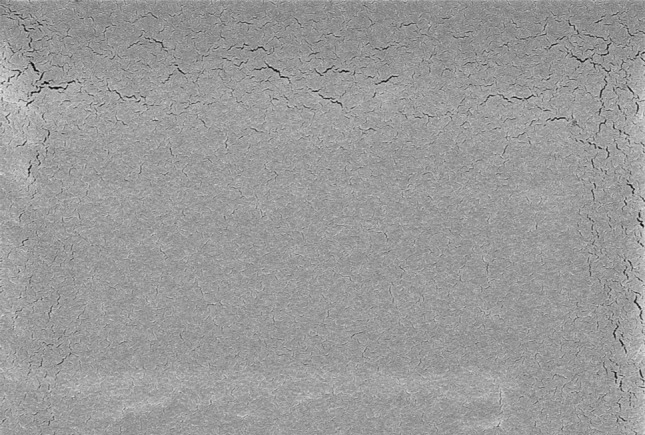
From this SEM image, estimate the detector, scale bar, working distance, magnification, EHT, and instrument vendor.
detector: InLens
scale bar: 1000 nm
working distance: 5.7 mm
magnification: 50 K X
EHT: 4 kV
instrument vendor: Zeiss